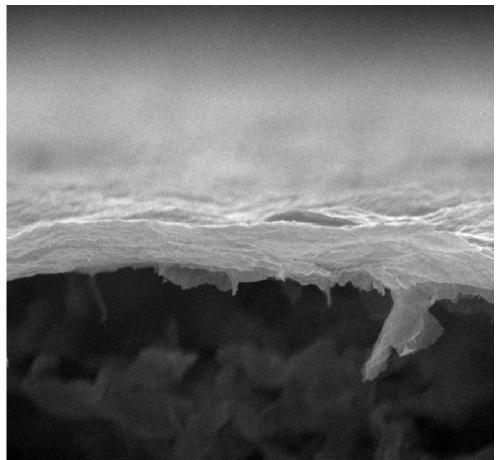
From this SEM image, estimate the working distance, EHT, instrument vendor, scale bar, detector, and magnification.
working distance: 10.7 mm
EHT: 15 kV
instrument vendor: JEOL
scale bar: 1000 nm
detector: LED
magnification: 16 K X